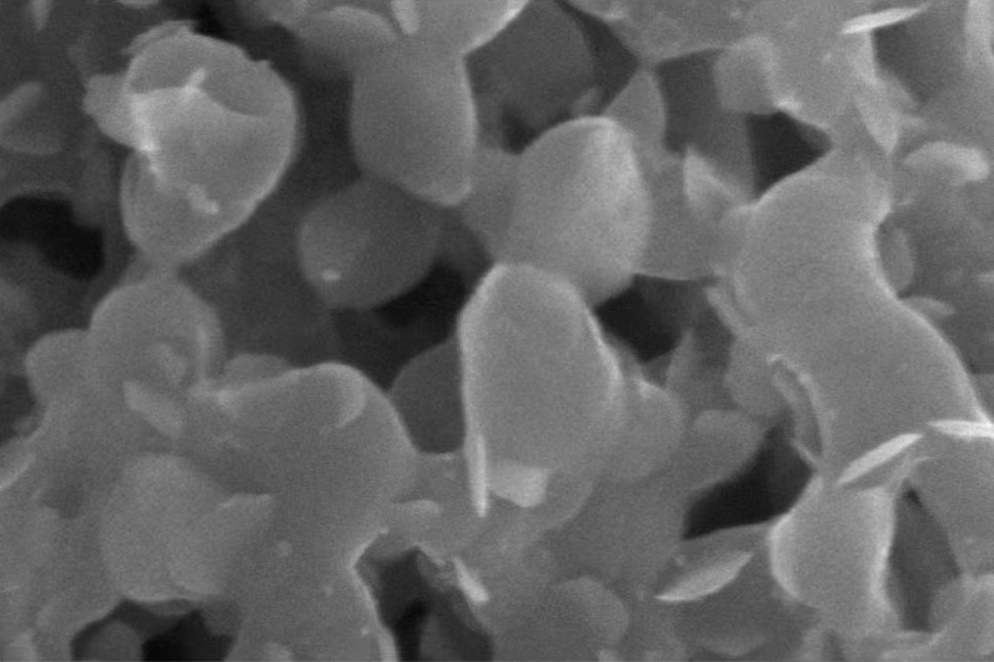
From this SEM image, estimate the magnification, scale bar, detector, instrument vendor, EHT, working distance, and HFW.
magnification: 100 K X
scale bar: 500 nm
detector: SE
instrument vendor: FEI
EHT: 15 kV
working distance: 4.9 mm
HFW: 2.07 µm